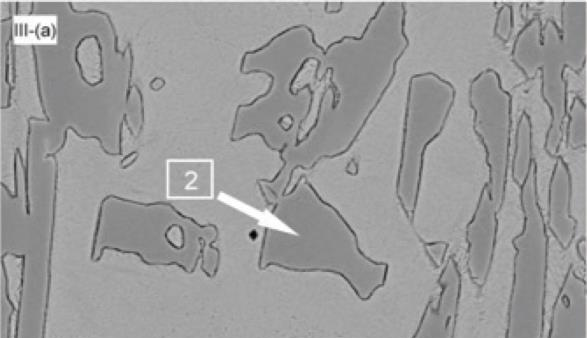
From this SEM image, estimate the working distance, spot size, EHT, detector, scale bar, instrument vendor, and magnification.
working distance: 9.9 mm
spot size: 5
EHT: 20 kV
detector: BSE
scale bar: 20000 nm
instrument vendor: FEI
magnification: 1 K X